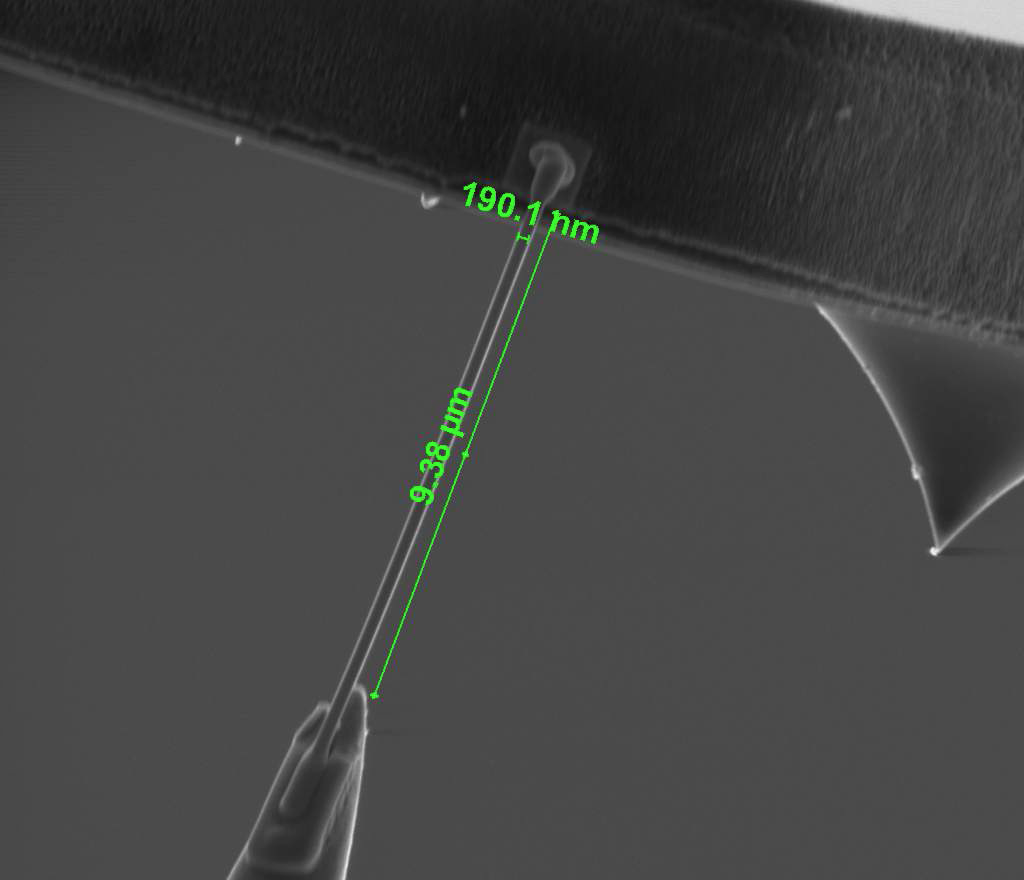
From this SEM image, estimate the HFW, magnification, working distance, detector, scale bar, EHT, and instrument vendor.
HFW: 18.6 µm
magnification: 8 K X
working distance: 10 mm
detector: SE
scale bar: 5000 nm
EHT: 5 kV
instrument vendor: FEI